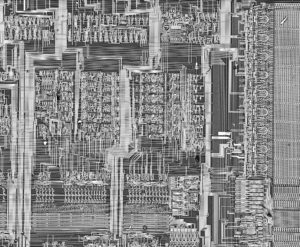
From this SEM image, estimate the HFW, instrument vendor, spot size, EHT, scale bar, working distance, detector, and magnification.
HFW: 1770 µm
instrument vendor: FEI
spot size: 5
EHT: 30 kV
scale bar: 500000 nm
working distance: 15.8 mm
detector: BSED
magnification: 0.072 K X